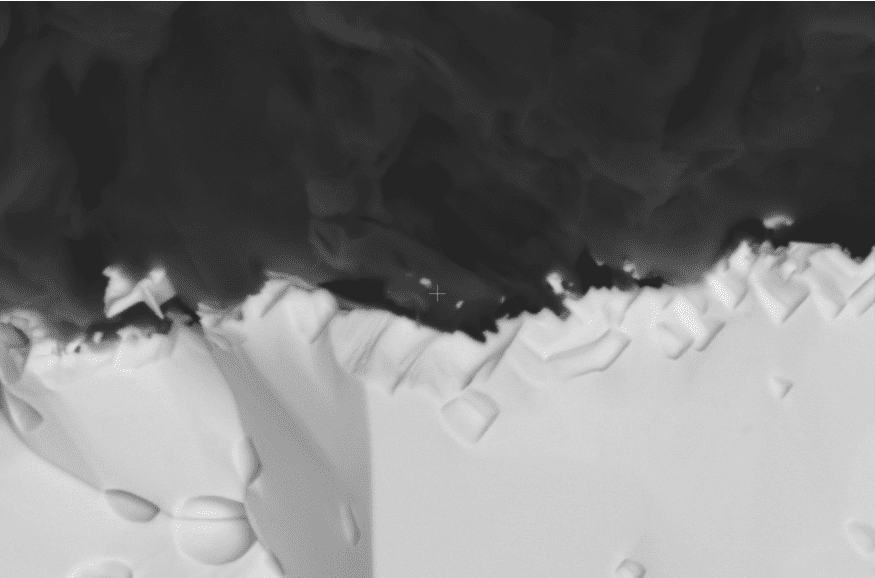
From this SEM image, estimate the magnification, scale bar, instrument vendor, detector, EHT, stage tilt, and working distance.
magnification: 10 K X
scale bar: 3000 nm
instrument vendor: FEI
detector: ABS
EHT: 10 kV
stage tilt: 0°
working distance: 10.6 mm